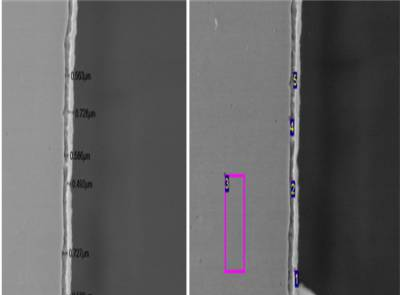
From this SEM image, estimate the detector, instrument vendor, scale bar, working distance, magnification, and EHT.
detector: LEI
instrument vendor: JEOL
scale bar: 1000 nm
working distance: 15.6 mm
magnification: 4 K X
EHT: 15 kV